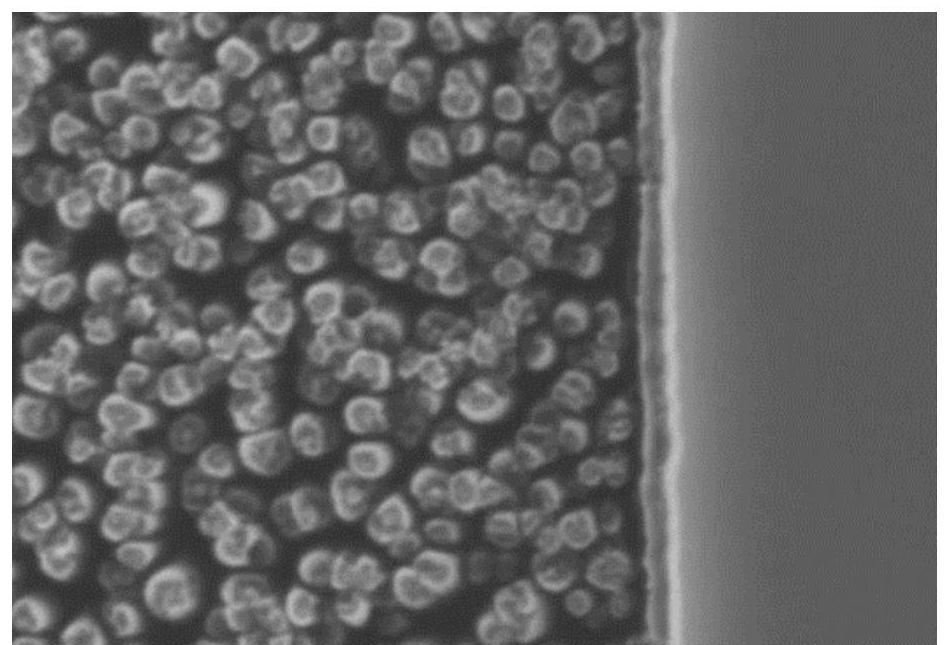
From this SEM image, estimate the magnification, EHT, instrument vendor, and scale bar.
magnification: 20 K X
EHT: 15 kV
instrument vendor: JEOL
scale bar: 1000 nm